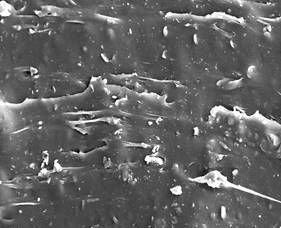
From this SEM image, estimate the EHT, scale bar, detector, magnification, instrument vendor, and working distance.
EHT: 20 kV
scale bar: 20000 nm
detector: SE1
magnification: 1 K X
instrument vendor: Zeiss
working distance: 6.5 mm